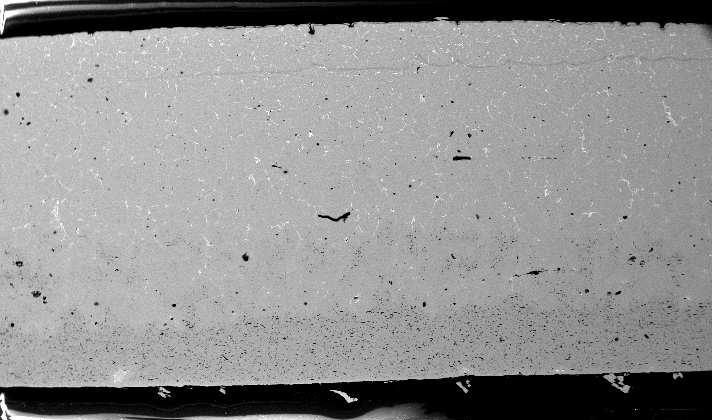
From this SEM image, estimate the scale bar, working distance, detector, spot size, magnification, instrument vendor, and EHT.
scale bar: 1000000 nm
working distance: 10 mm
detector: BSE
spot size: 4.8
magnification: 0.05 K X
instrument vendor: FEI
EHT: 15 kV